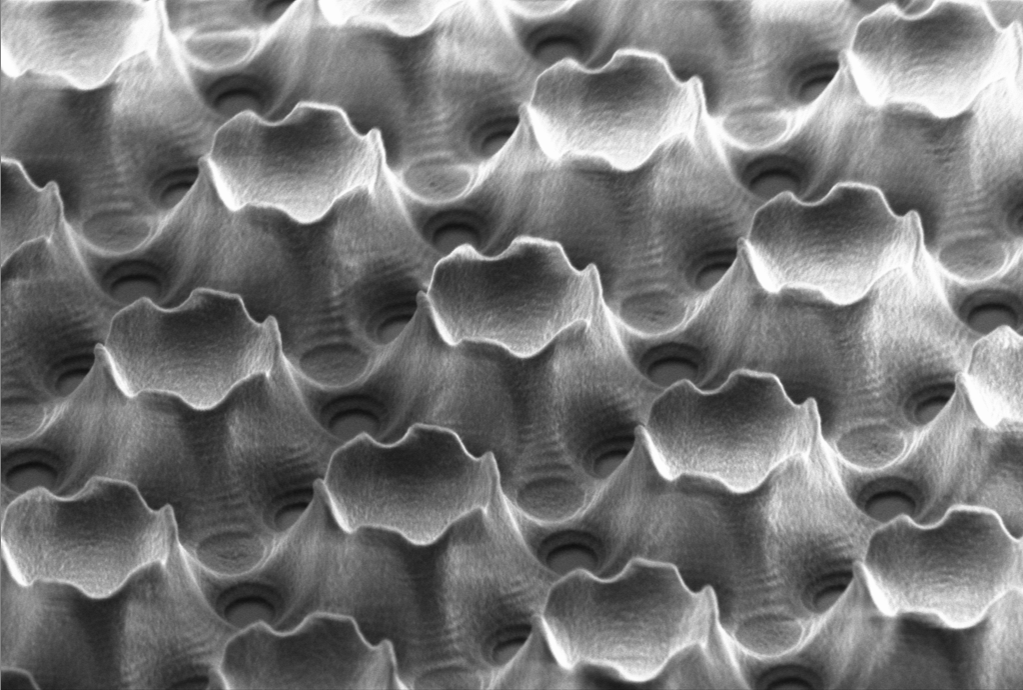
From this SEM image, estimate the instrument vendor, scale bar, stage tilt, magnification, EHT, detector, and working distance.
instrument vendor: Zeiss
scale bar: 2000 nm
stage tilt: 53°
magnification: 7.26 K X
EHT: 1.3 kV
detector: SE2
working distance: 6.8 mm